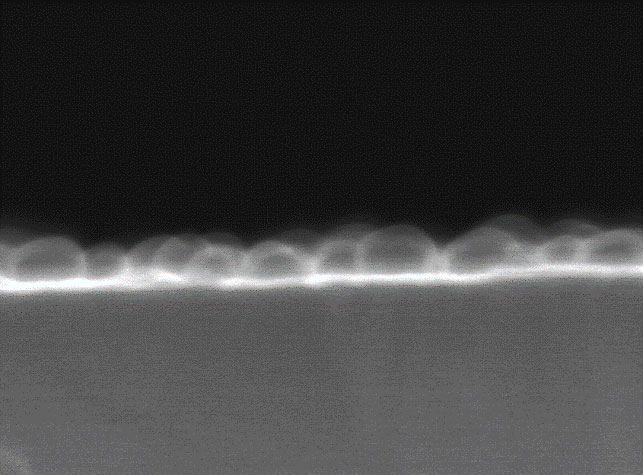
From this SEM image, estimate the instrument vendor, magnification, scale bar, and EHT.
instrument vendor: JEOL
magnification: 300 K X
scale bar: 100 nm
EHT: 20 kV